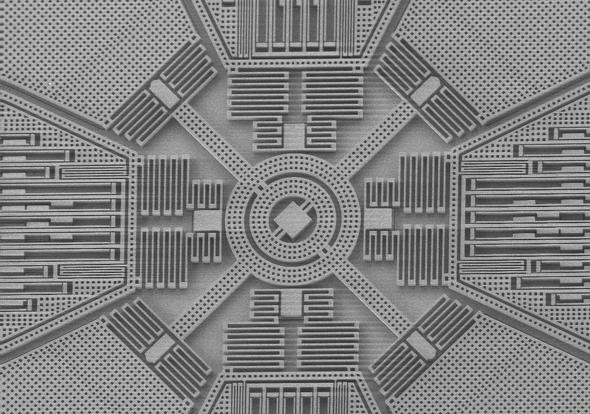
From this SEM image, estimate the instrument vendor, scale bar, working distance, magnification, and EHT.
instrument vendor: Zeiss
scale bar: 200000 nm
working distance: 4 mm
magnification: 0.135 K X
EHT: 3 kV